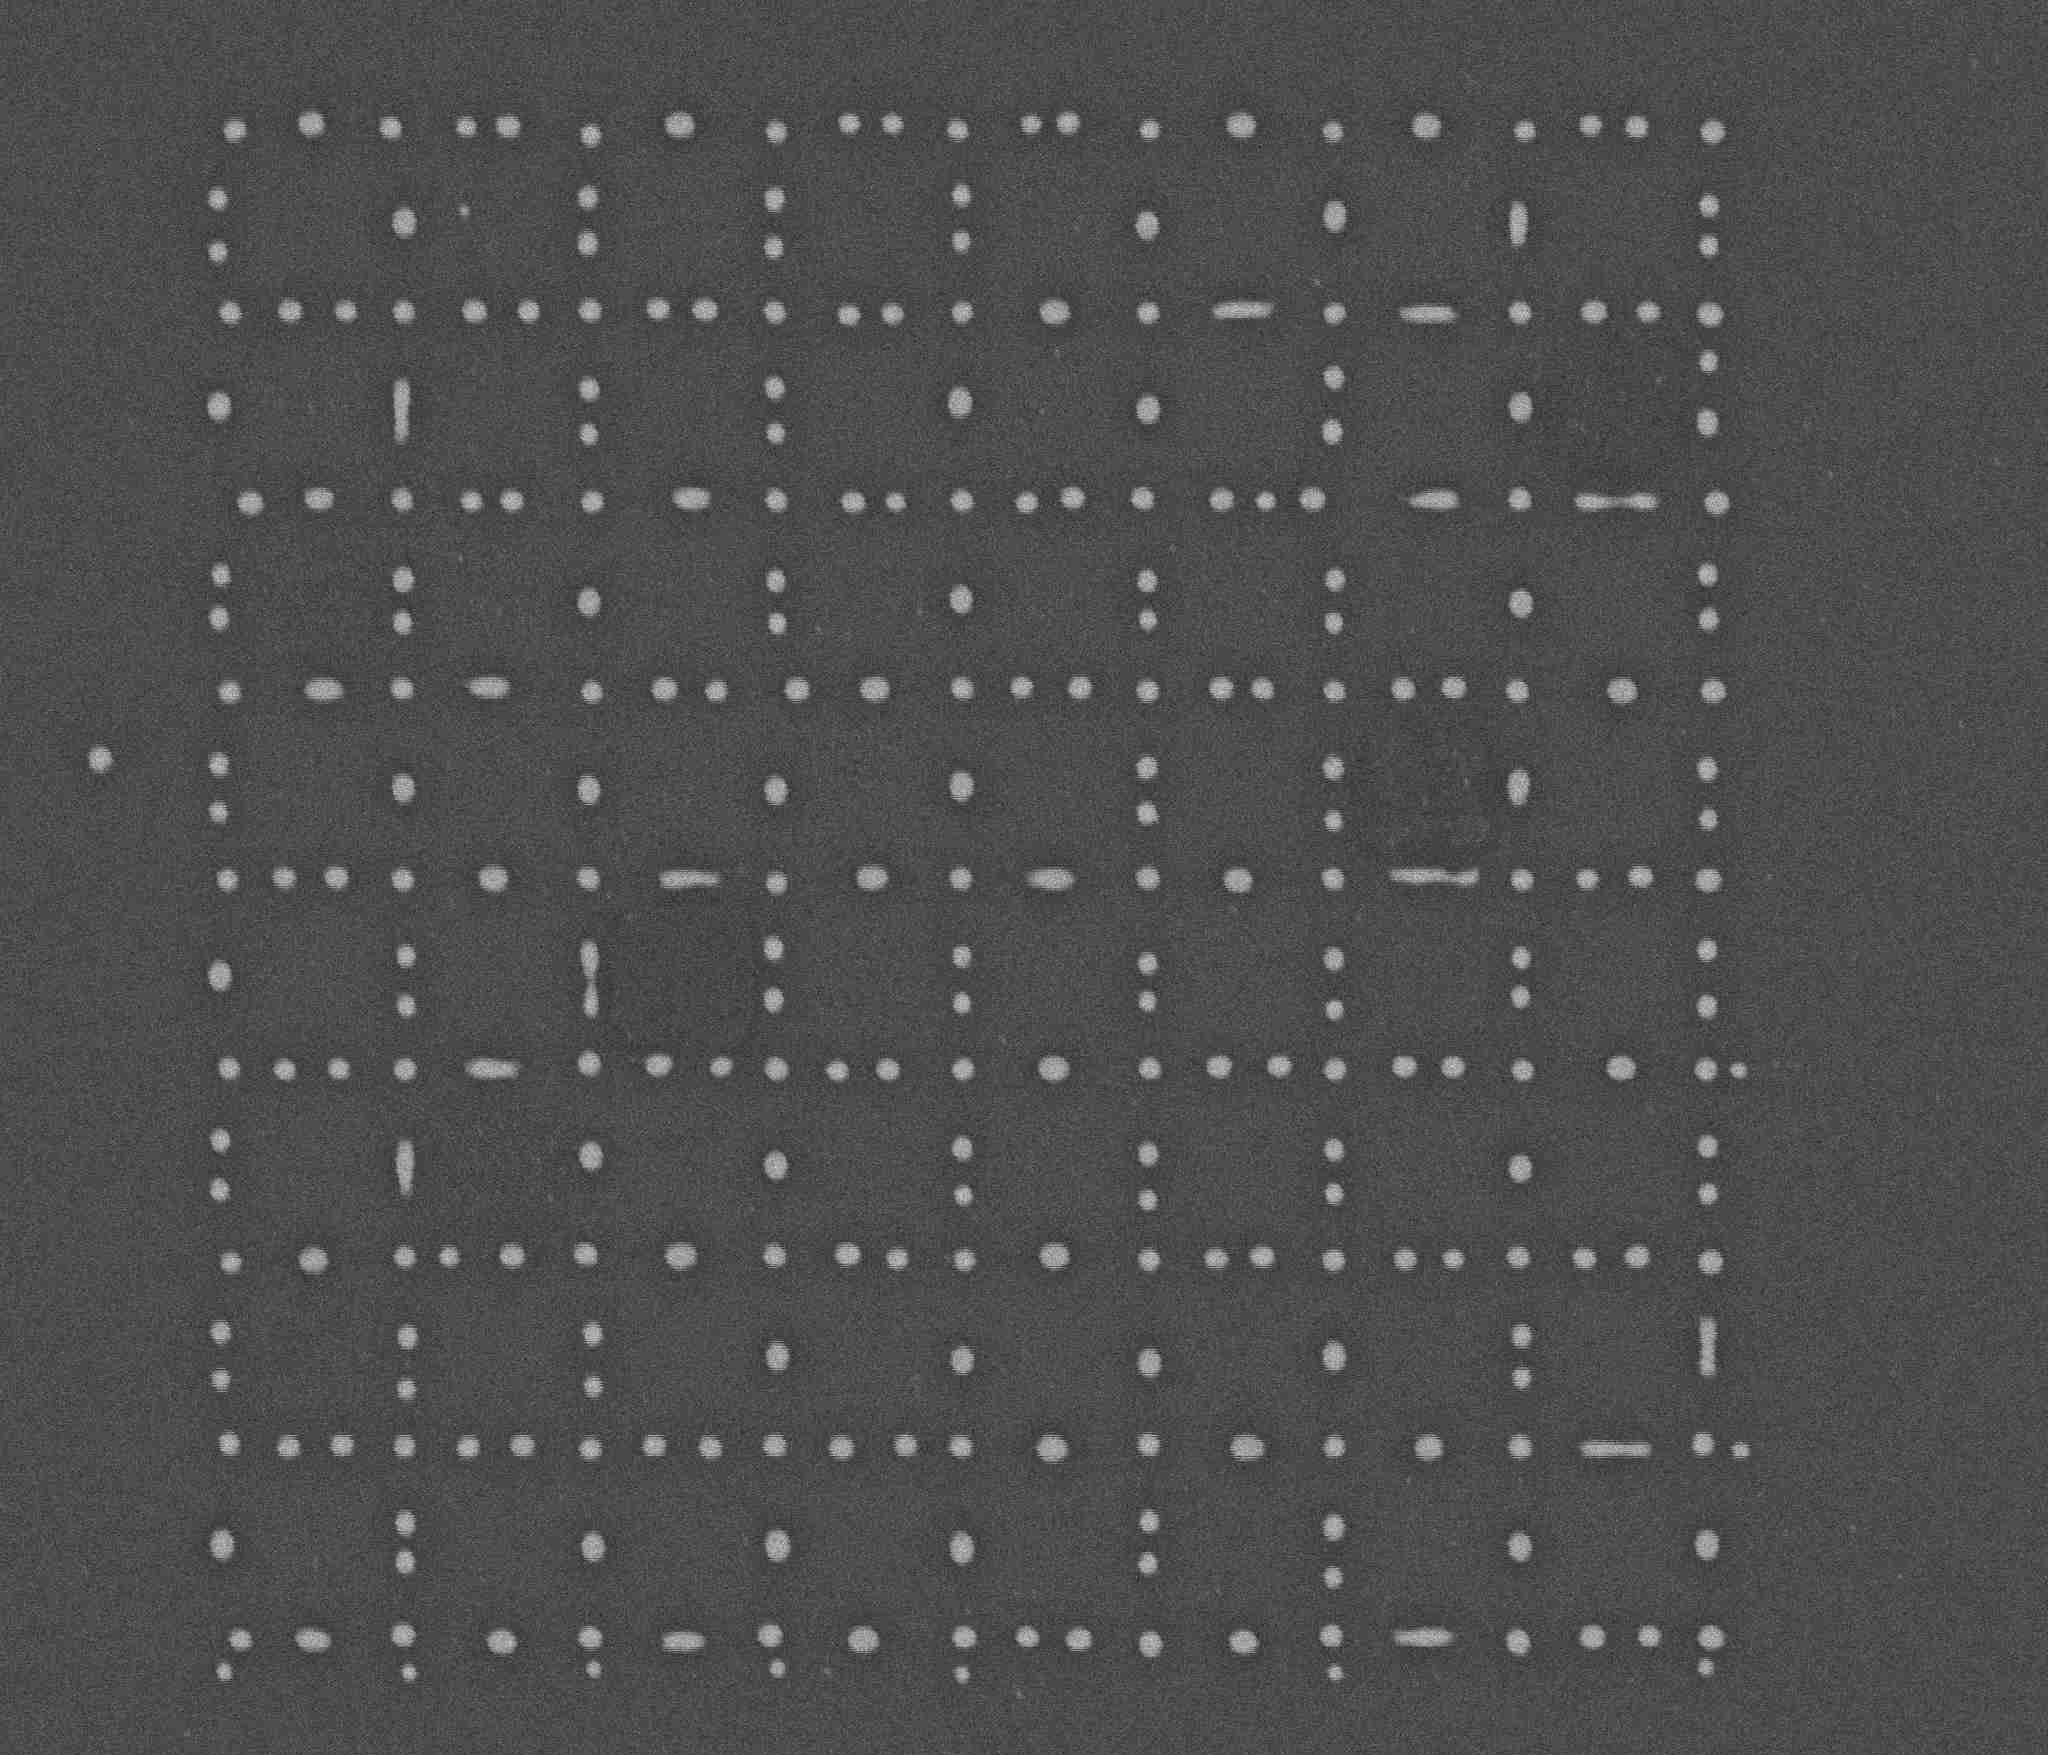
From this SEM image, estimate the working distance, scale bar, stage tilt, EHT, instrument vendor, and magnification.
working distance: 5.2 mm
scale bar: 5000 nm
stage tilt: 0°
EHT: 5 kV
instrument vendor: FEI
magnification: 10 K X